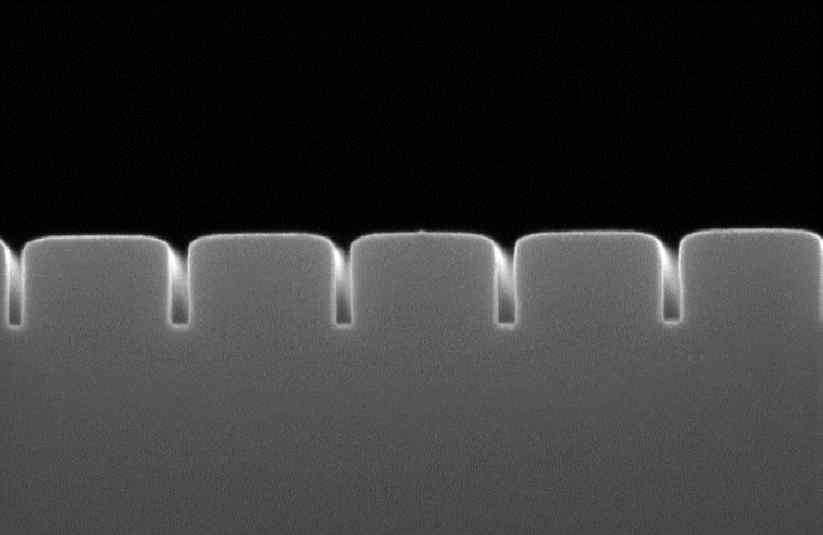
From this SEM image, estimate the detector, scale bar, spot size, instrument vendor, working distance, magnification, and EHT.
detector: TLD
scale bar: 200 nm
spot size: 3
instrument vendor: FEI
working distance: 5.2 mm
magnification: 200 K X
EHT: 10 kV